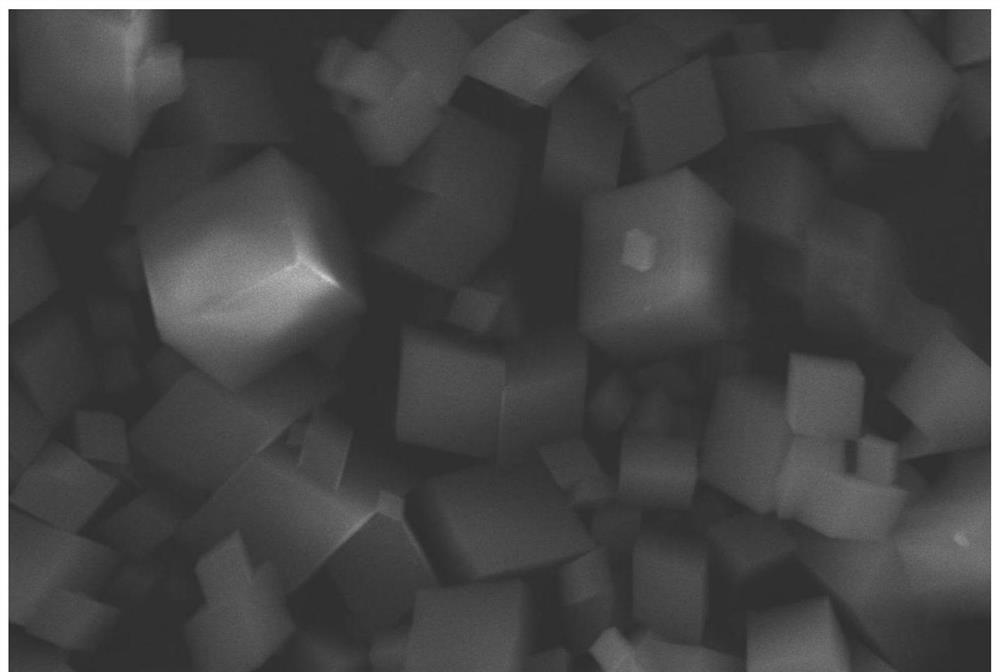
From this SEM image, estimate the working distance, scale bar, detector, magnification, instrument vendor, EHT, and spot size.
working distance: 12 mm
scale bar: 5000 nm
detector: SEI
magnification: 3 K X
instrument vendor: JEOL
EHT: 20 kV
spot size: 36